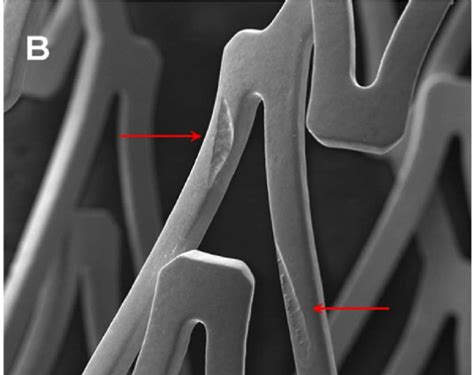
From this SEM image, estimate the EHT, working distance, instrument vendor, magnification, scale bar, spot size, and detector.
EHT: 30 kV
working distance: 8.4 mm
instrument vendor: FEI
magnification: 0.182 K X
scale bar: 500000 nm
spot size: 4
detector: ETD